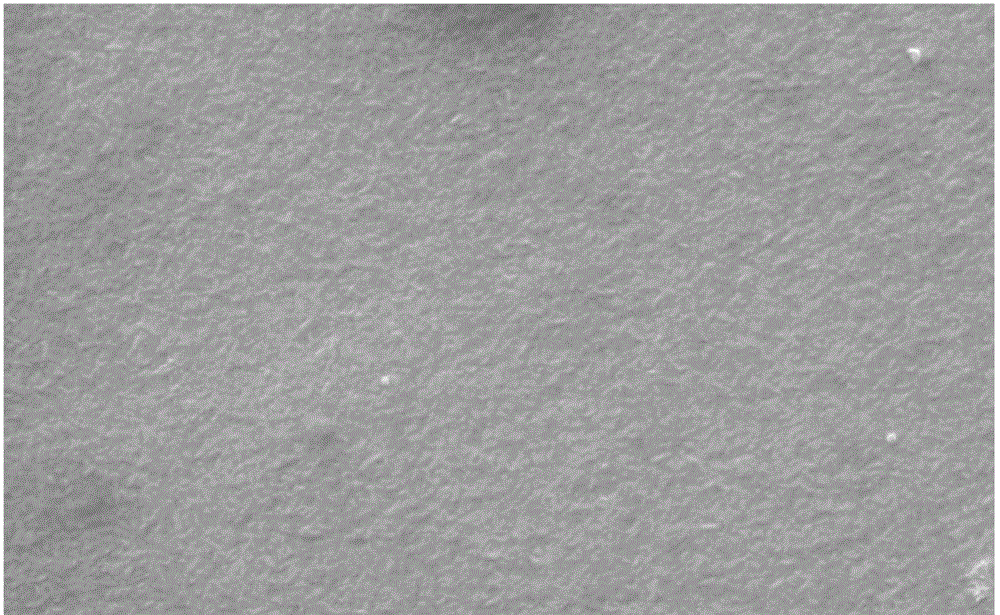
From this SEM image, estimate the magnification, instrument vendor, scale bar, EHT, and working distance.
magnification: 10 K X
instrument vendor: Zeiss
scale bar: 2000 nm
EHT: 15 kV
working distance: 12.5 mm